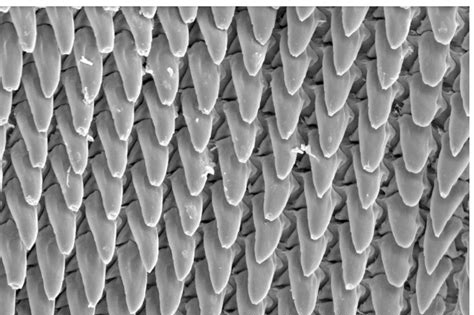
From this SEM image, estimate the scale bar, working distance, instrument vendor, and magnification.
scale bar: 100000 nm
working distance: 16.4 mm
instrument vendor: JEOL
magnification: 0.5 K X